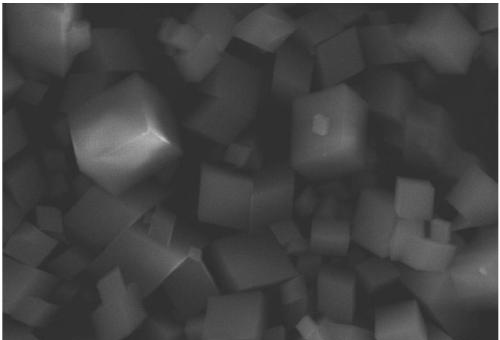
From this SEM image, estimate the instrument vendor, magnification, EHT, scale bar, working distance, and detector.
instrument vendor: JEOL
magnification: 3 K X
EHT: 20 kV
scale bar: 5000 nm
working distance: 12 mm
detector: SEI